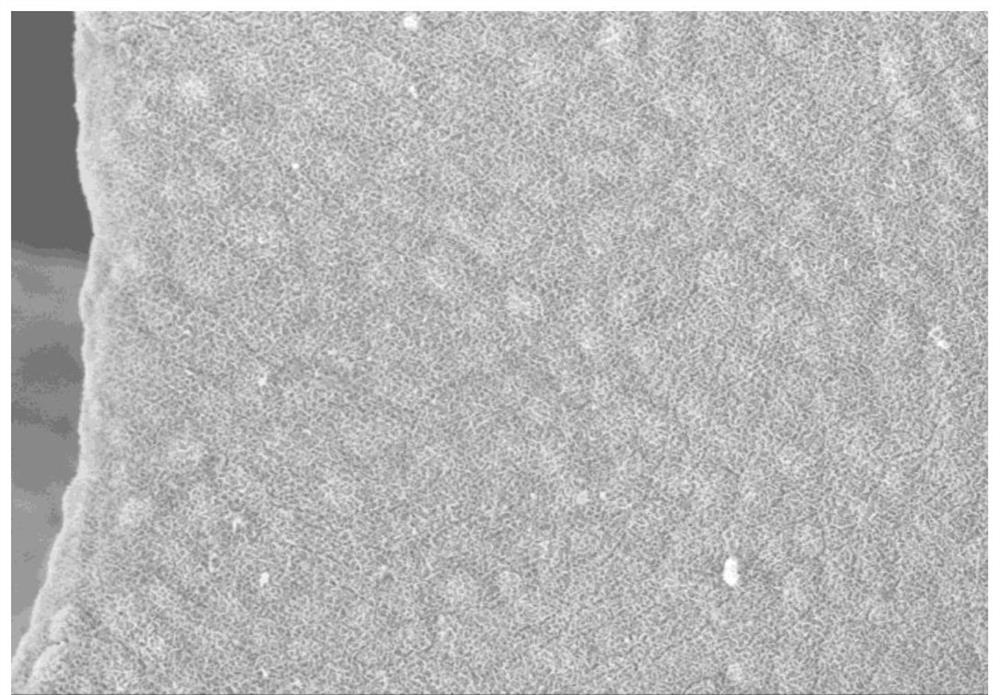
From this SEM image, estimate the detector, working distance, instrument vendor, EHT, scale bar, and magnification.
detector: SE(U)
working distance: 9.7 mm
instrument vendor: Hitachi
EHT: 5 kV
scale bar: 20000 nm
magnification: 2 K X